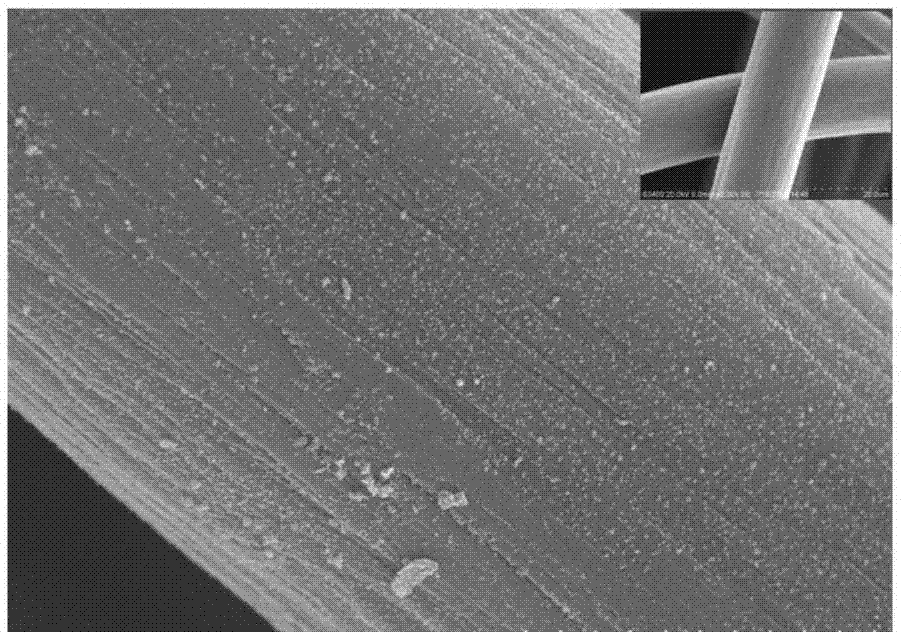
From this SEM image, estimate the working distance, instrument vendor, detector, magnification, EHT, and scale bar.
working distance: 7.5 mm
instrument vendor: Hitachi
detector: SE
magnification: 5 K X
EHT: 20 kV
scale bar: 10000 nm